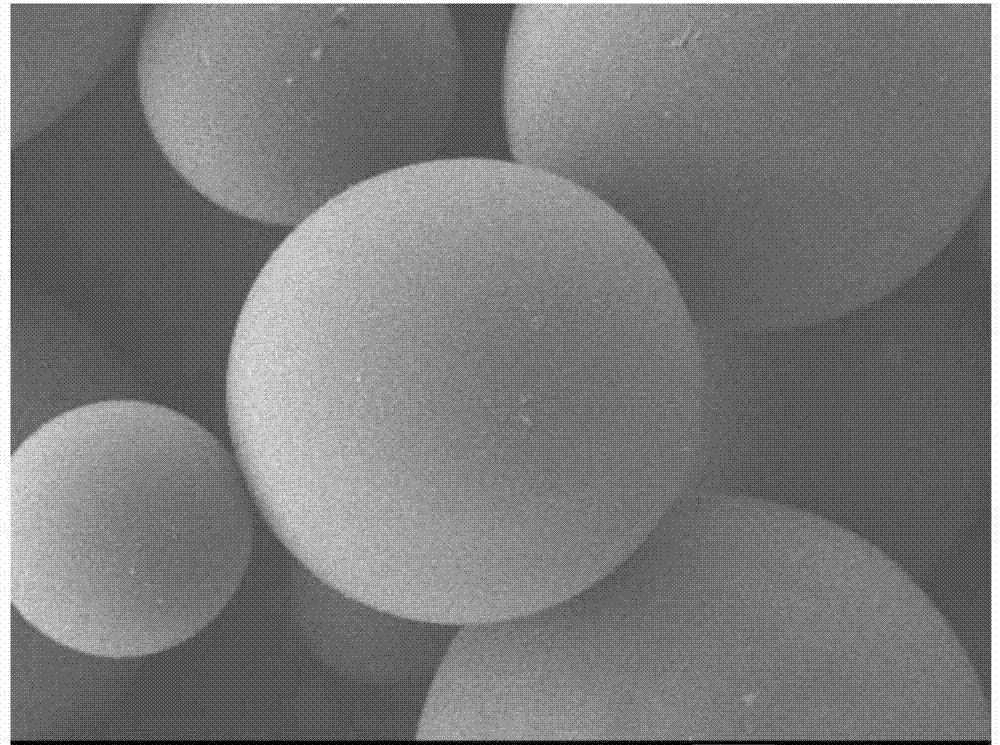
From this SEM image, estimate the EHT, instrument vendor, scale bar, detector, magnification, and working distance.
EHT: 1 kV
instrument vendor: JEOL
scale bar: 10000 nm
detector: LEI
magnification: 1 K X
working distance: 8 mm